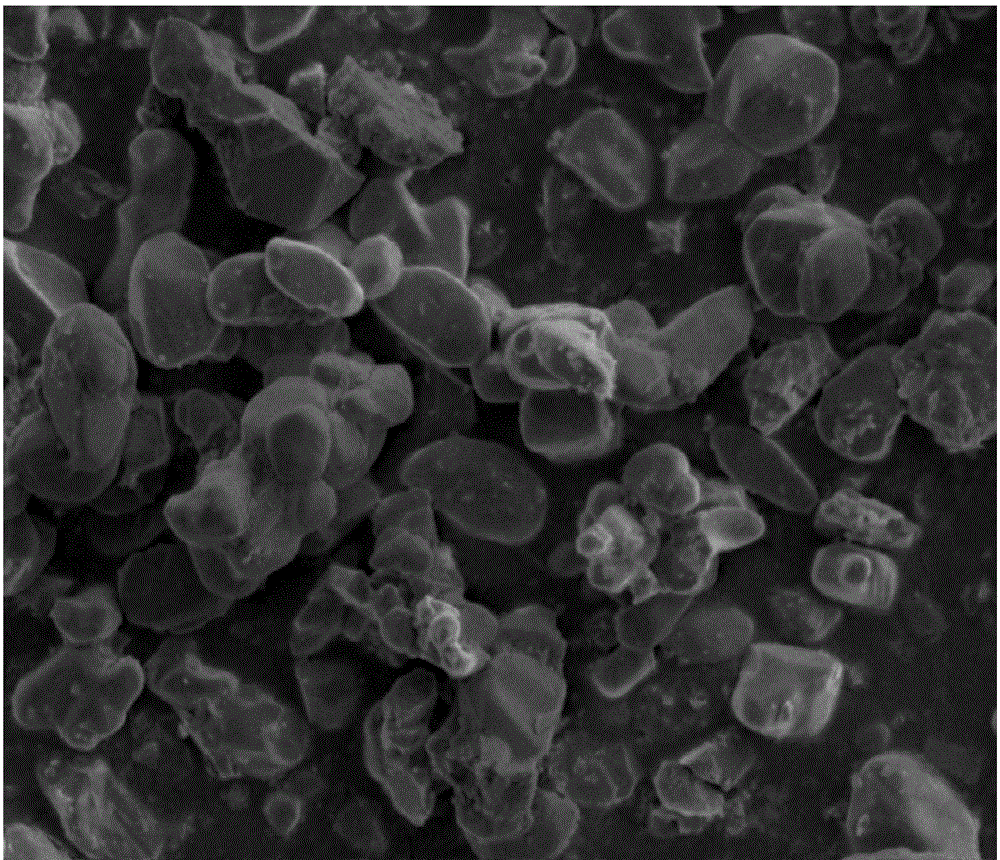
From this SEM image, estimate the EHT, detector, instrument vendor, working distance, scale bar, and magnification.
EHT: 5 kV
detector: ETD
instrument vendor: FEI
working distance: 4.8 mm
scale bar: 5000 nm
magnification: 10 K X